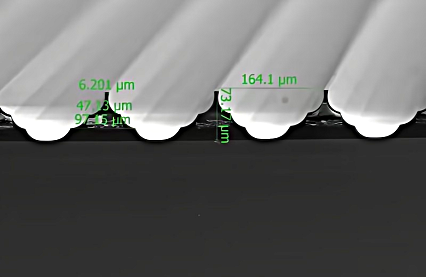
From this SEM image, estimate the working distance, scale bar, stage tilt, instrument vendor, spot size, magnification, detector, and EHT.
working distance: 10.4 mm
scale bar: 200000 nm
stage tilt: -2°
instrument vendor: FEI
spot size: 10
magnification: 0.2 K X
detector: ETD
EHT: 20 kV